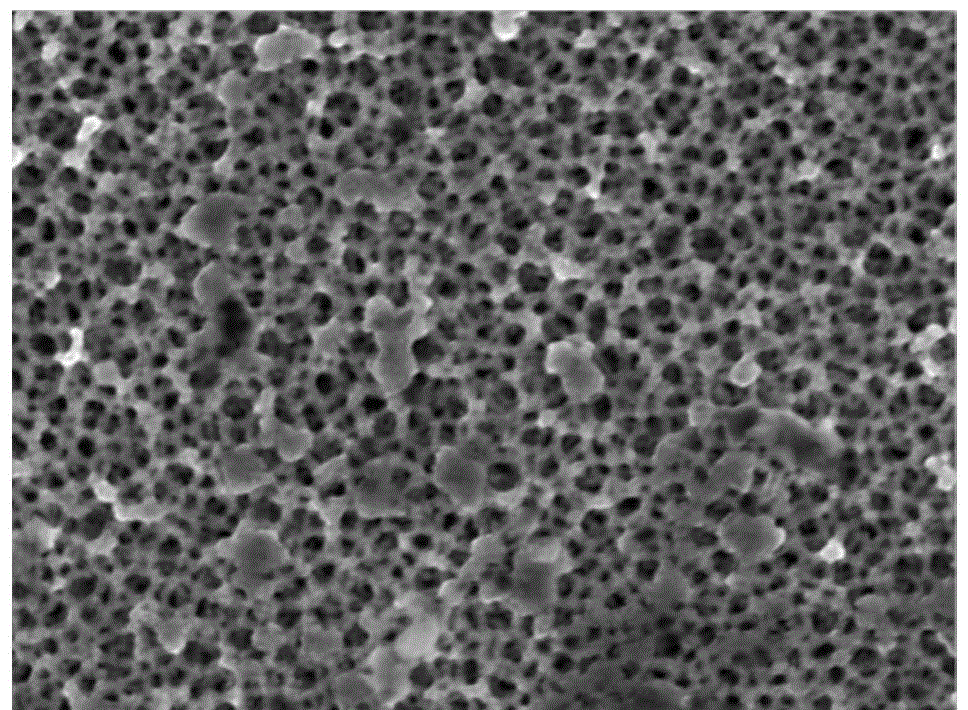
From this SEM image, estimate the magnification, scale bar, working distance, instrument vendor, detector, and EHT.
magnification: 30 K X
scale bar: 100 nm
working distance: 6.9 mm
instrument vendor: JEOL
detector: SEI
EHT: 5 kV